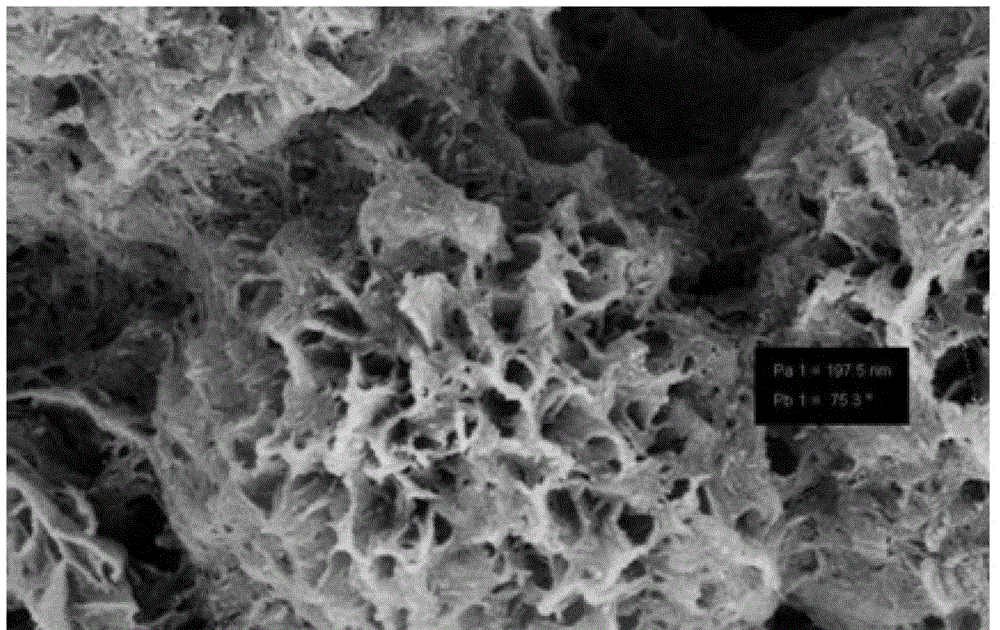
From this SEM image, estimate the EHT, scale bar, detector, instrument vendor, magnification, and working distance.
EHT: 5 kV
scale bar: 200 nm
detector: SE2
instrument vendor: Zeiss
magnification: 100 K X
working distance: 6.9 mm